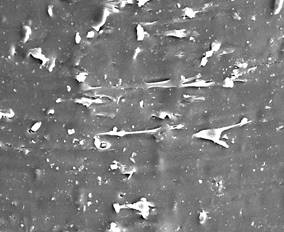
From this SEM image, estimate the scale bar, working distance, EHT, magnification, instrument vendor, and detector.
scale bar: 20000 nm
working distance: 8.5 mm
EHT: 20 kV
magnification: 1 K X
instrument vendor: Zeiss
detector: SE1